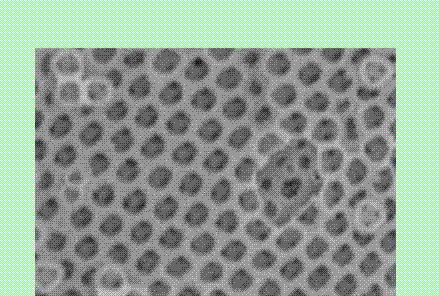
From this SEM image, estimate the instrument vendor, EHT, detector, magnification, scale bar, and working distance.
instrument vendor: Hitachi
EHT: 15 kV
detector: SE(U)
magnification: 50 K X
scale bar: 1000 nm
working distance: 6.6 mm